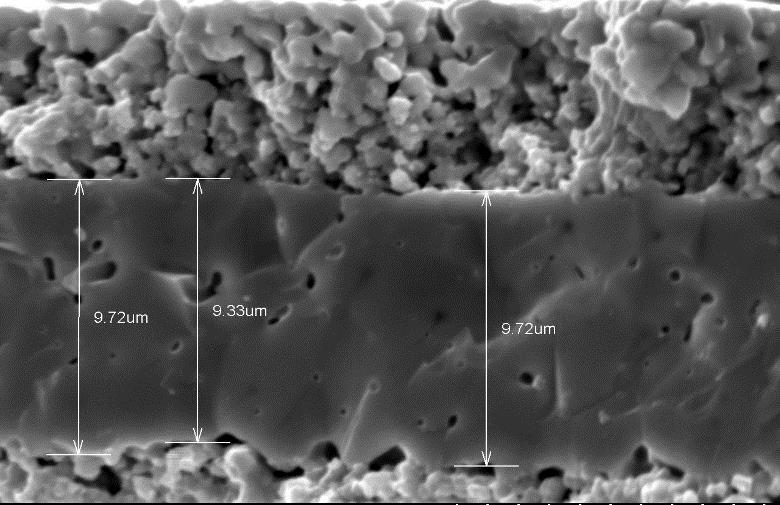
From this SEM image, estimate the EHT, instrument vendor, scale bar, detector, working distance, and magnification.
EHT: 20 kV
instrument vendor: Hitachi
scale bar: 10000 nm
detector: SE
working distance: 9.7 mm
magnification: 5 K X